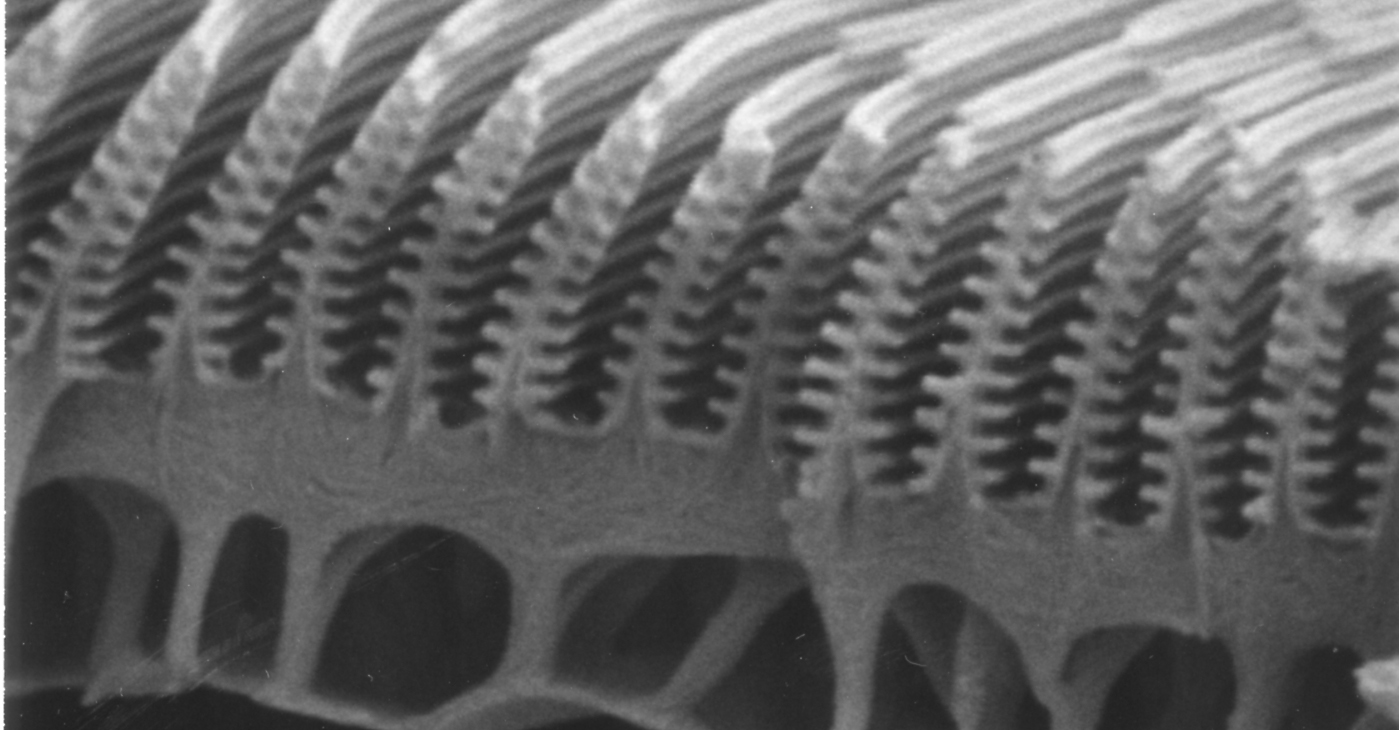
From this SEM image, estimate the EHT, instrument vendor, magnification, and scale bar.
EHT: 5 kV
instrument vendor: JEOL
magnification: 15 K X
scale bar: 1000 nm